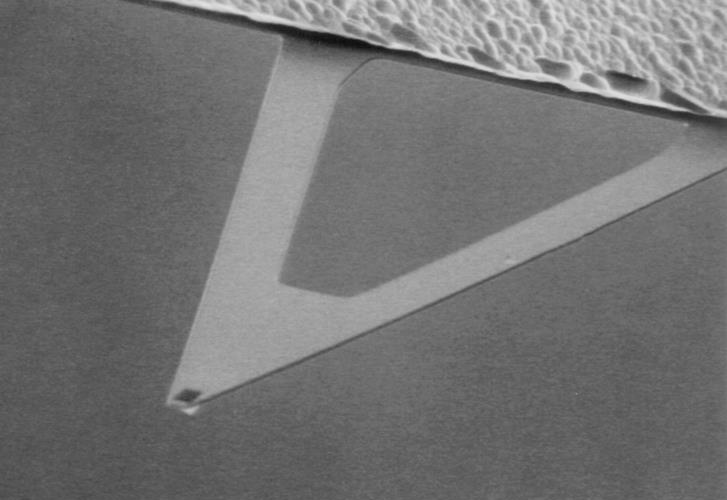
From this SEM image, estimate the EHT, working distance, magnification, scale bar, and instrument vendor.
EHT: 5 kV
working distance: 18 mm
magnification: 0.8 K X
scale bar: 10000 nm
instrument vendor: JEOL